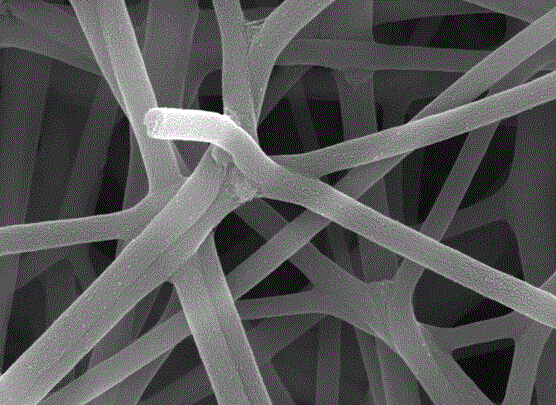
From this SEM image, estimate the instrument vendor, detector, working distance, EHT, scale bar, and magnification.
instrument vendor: JEOL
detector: SEI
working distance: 8.8 mm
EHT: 10 kV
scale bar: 100 nm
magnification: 30 K X